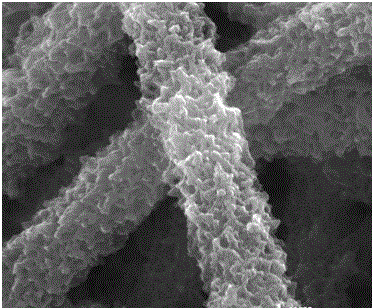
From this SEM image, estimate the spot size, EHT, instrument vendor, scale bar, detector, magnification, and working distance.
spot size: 3.5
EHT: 30 kV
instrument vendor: FEI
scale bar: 1000 nm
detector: ETD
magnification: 80 K X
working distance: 8.9 mm